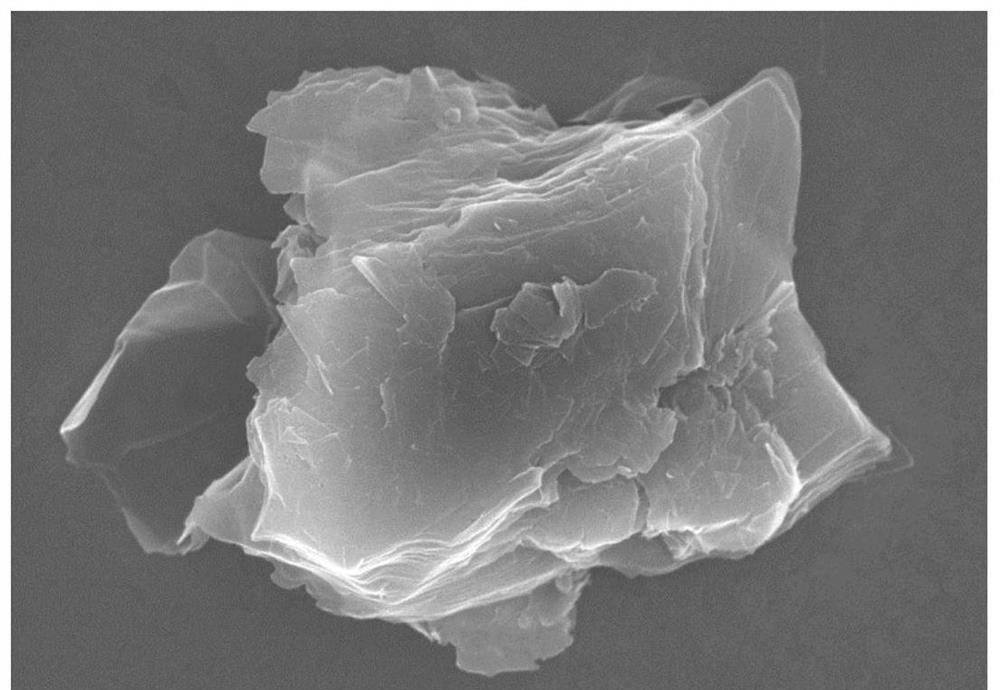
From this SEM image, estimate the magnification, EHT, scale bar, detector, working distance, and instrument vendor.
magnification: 20 K X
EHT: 10 kV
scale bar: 2000 nm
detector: SE(M)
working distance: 9.1 mm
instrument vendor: Hitachi